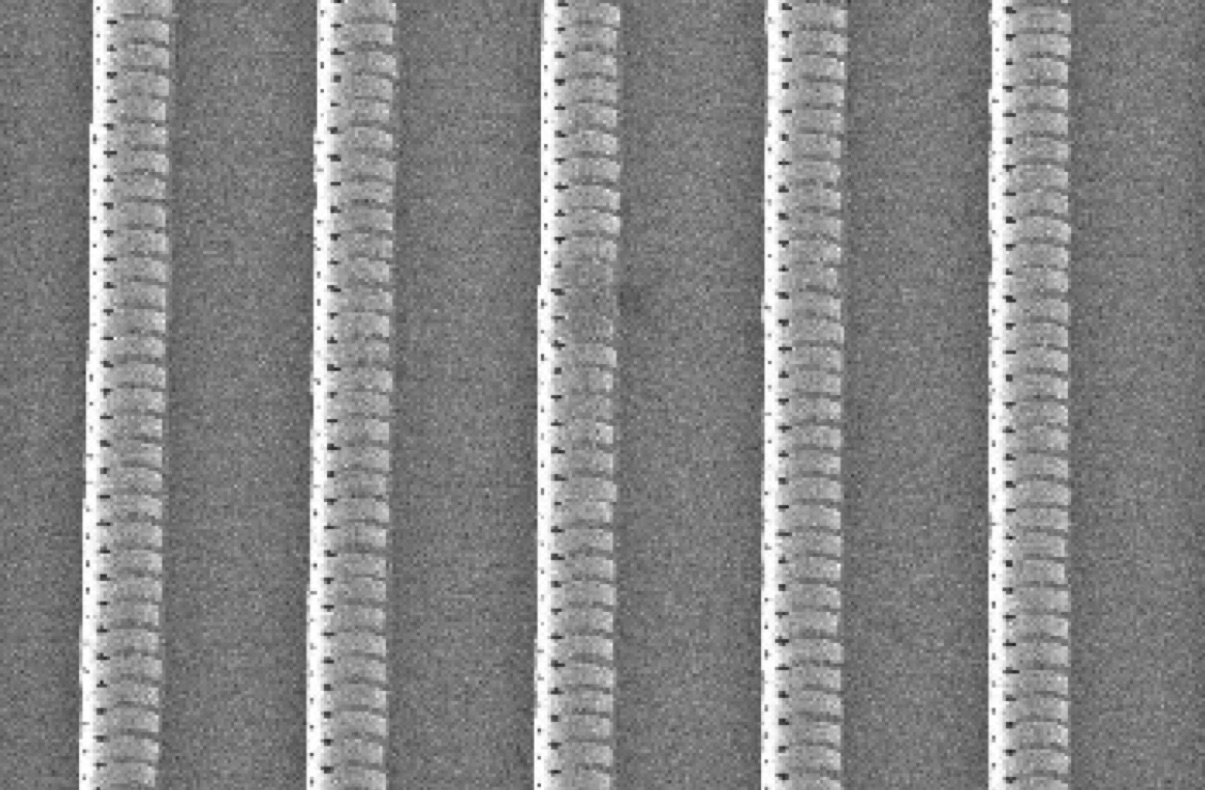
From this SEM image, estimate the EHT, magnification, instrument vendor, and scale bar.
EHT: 3 kV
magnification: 0.1 K X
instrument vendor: JEOL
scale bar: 100000 nm